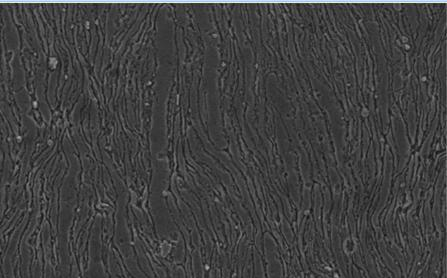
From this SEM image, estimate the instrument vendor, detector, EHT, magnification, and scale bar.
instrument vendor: JEOL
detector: SEI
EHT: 15 kV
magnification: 0.5 K X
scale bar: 50000 nm